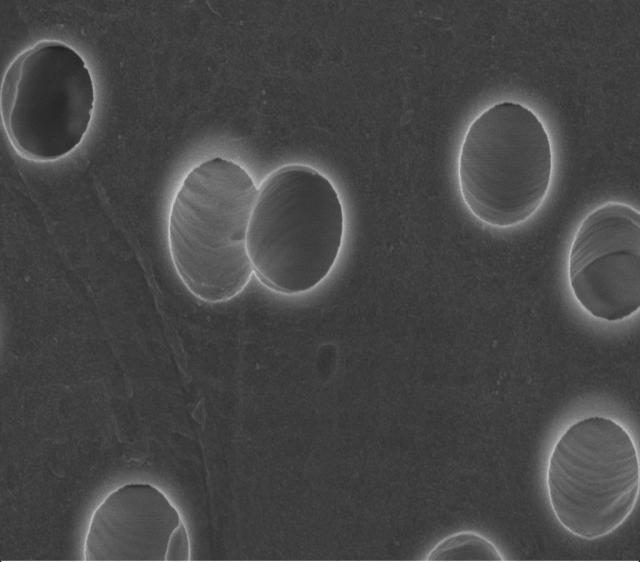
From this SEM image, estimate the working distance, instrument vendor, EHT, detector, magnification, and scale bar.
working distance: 3 mm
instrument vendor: Zeiss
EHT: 3 kV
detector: InLens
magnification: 65 K X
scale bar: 200 nm